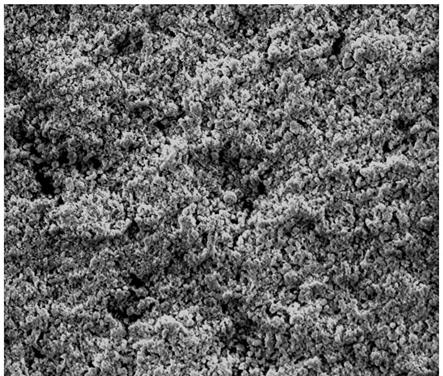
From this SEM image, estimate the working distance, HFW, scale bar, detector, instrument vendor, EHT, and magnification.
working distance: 11.1 mm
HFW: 254 µm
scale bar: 100000 nm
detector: ETD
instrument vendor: FEI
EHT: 5 kV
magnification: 0.5 K X